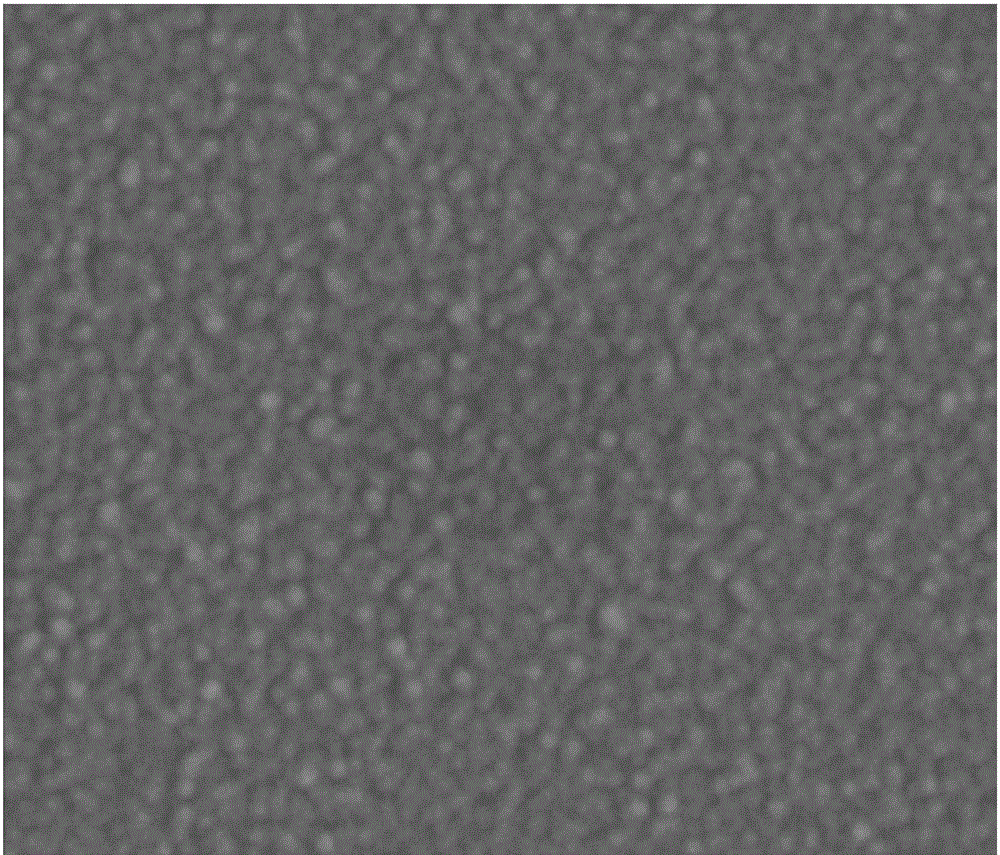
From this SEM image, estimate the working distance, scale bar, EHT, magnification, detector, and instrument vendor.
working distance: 5.3 mm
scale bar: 2000 nm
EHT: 5 kV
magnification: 40 K X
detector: ETD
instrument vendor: FEI